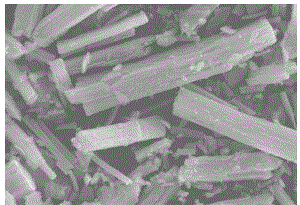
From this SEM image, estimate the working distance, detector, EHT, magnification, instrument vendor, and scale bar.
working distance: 10.2 mm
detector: SE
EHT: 30 kV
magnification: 1.6 K X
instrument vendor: Hitachi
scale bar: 30000 nm